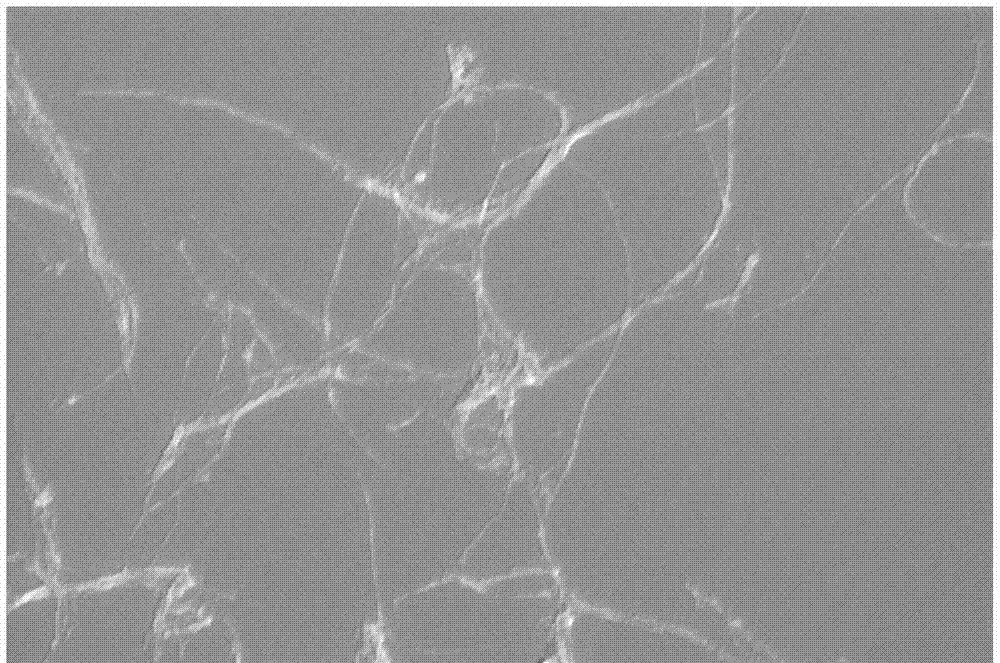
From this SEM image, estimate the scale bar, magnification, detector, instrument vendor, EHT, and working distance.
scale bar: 200 nm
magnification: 30 K X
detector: InLens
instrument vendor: Zeiss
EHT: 5 kV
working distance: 4.9 mm